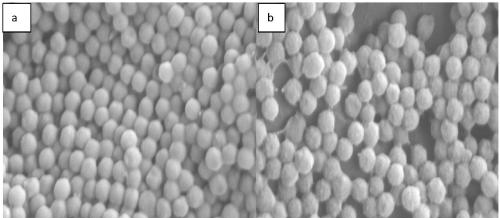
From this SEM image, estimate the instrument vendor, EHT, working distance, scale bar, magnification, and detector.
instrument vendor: Zeiss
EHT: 5 kV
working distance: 8.7 mm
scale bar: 200 nm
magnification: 50 K X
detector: SE2